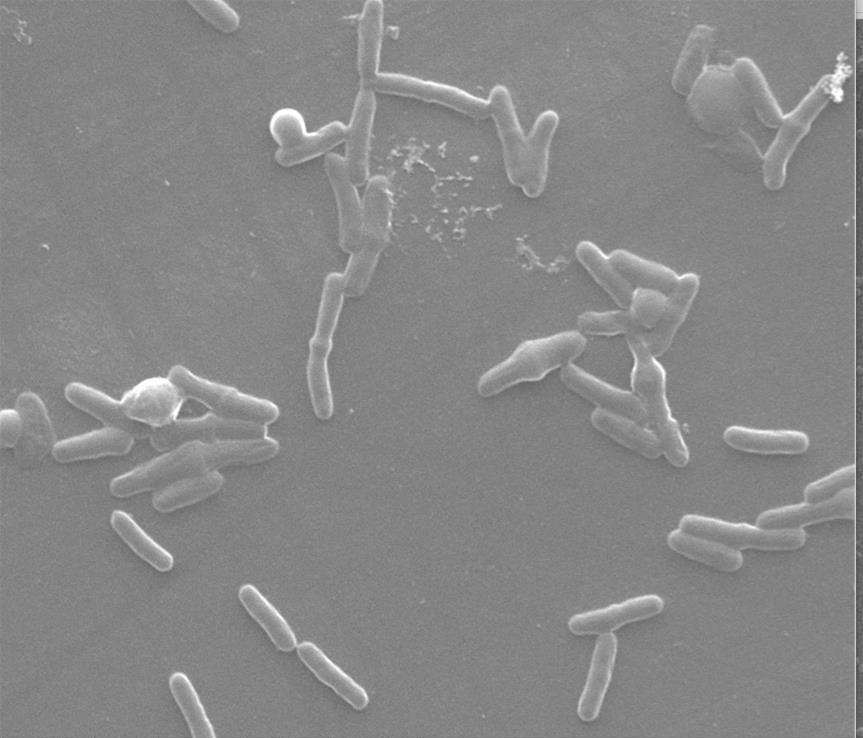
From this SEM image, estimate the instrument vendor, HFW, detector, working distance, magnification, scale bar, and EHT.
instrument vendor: FEI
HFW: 32 µm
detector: SE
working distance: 9.7 mm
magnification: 8 K X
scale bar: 10000 nm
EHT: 30 kV